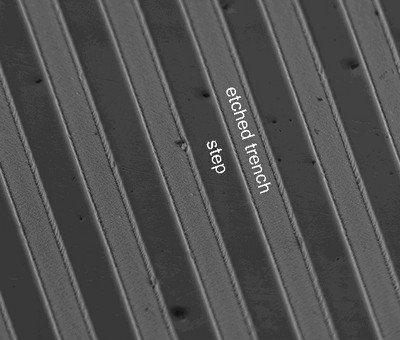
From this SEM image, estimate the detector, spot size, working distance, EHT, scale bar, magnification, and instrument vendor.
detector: TLD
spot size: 3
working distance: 6.3 mm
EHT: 5 kV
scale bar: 4000 nm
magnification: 16.839 K X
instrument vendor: FEI